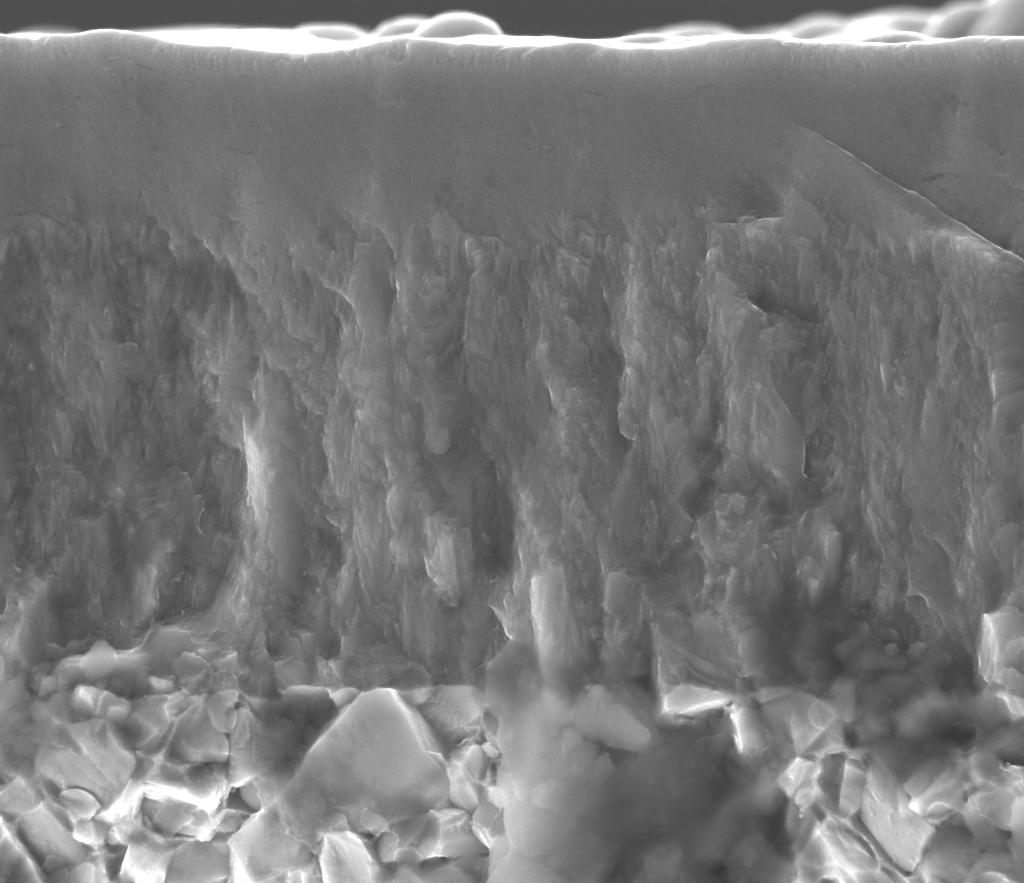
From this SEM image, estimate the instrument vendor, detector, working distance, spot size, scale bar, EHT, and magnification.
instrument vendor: FEI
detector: ETD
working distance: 8.9 mm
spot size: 3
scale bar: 4000 nm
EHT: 10 kV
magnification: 36 K X